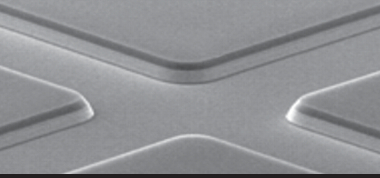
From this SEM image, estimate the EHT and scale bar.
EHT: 20 kV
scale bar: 10000 nm